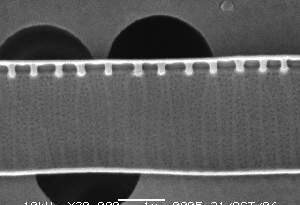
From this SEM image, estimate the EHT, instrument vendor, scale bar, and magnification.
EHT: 10 kV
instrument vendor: JEOL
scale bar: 1000 nm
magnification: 20 K X